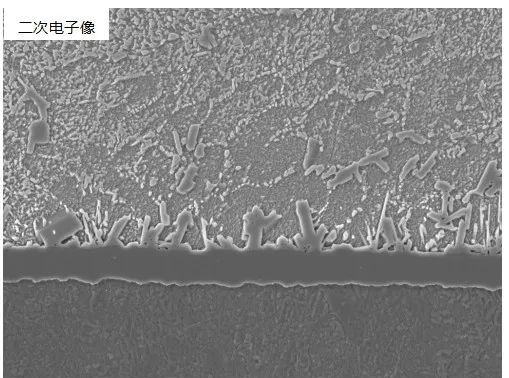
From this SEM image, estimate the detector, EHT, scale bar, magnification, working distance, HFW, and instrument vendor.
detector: SE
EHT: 20 kV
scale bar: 20000 nm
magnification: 3.23 K X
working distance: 10.01 mm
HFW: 85.8 µm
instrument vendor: Tescan